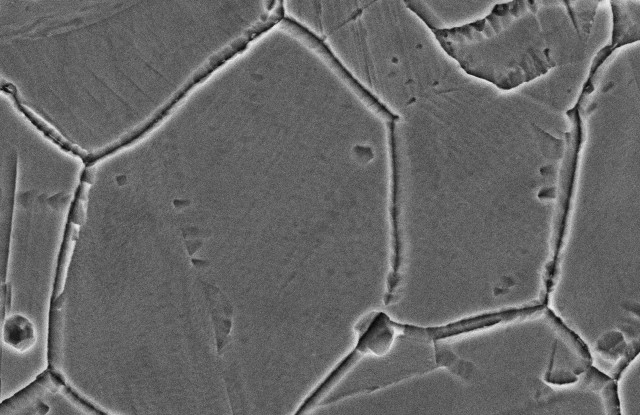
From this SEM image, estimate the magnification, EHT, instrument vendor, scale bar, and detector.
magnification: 3 K X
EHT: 15 kV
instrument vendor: JEOL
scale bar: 10000 nm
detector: SEI